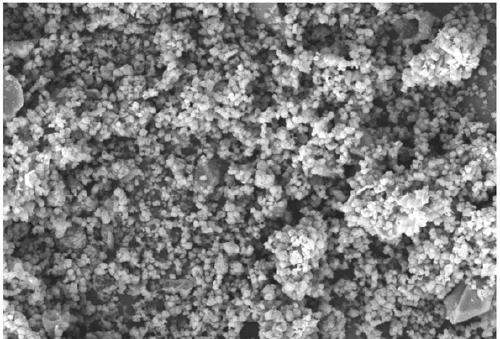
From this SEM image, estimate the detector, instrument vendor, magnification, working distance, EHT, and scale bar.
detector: SE2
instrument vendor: Zeiss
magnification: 5 K X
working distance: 9 mm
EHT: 10 kV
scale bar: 2000 nm